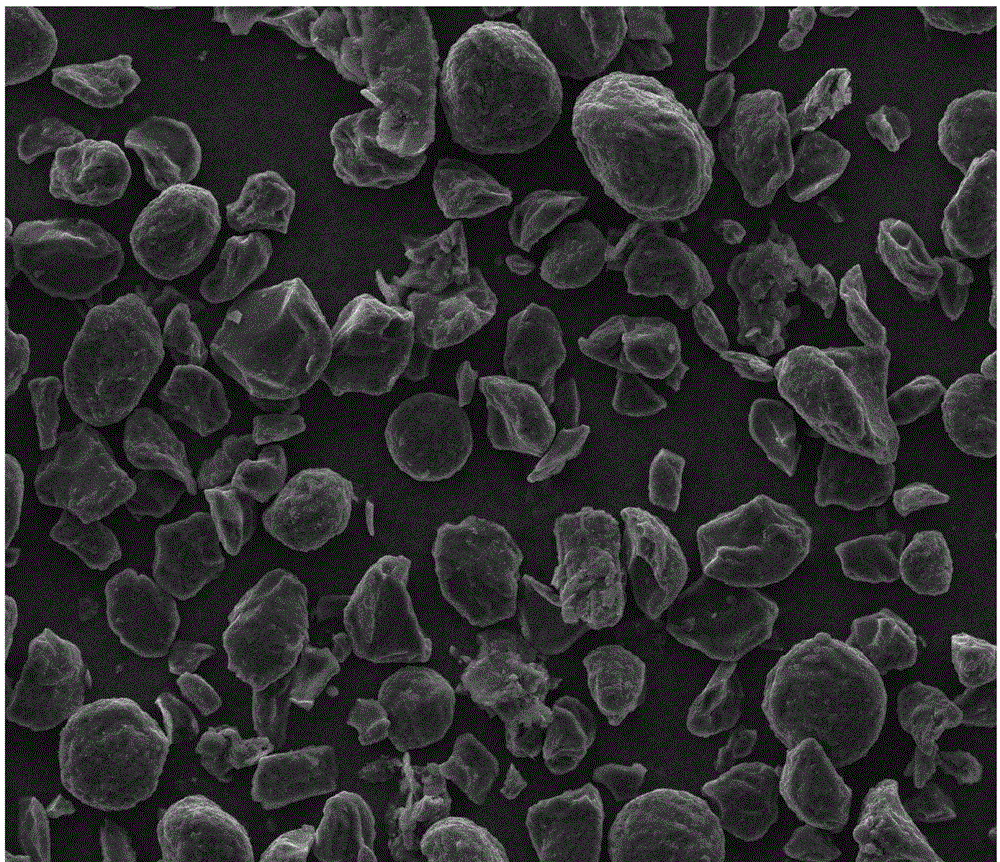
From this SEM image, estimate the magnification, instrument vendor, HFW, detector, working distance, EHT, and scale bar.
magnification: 0.5 K X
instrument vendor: FEI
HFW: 270 µm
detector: ETD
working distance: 10.6 mm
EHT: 15 kV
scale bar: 50000 nm